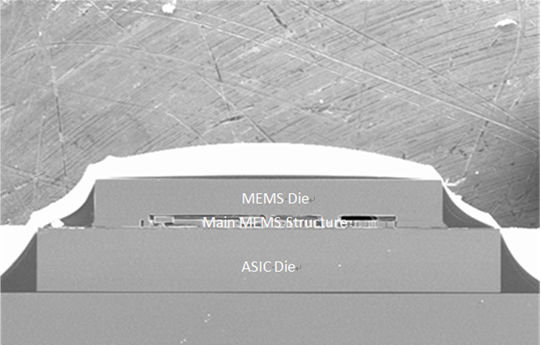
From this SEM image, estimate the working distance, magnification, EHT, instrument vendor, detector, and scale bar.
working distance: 11.5 mm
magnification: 0.05 K X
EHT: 20 kV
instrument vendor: Hitachi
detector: SE(M)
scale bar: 1000000 nm